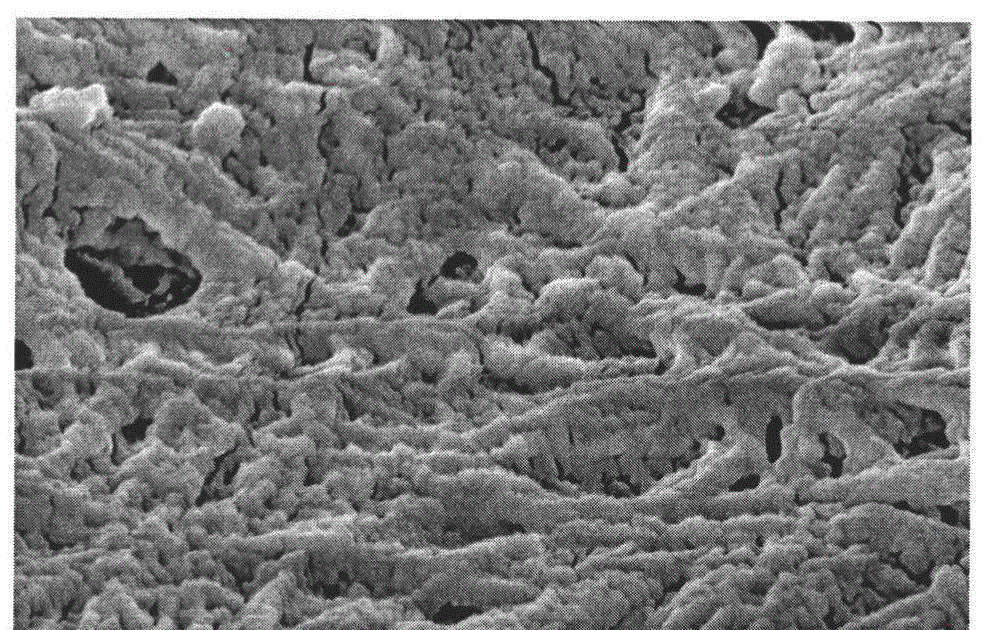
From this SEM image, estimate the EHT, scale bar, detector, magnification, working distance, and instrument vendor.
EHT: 5 kV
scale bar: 500 nm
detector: SE(M)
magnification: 70 K X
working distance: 4.9 mm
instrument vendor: Hitachi